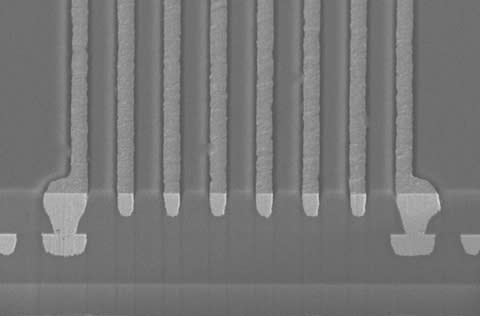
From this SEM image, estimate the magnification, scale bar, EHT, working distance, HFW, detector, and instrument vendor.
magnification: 2 K X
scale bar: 30000 nm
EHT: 15 kV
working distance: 4 mm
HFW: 104 µm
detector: TLD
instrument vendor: FEI